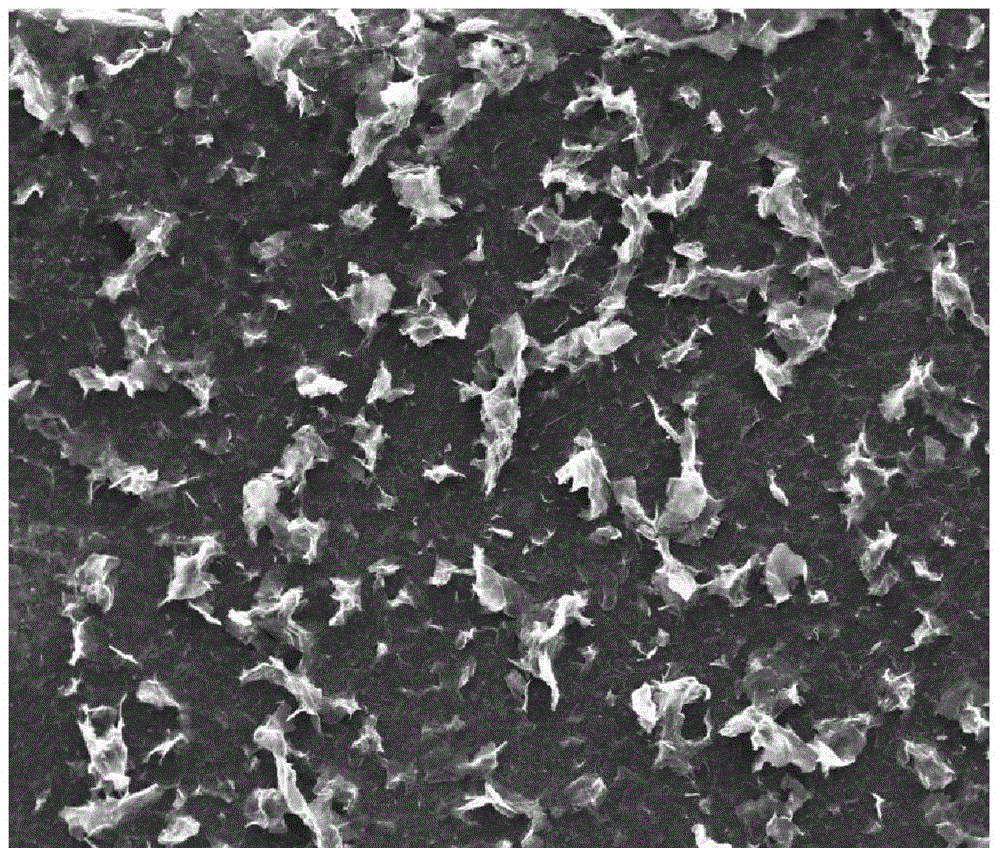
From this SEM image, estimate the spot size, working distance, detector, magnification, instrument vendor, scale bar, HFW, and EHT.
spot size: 3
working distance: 11.3 mm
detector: ETD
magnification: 0.25 K X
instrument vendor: FEI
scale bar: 500000 nm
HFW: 1190 µm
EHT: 20 kV